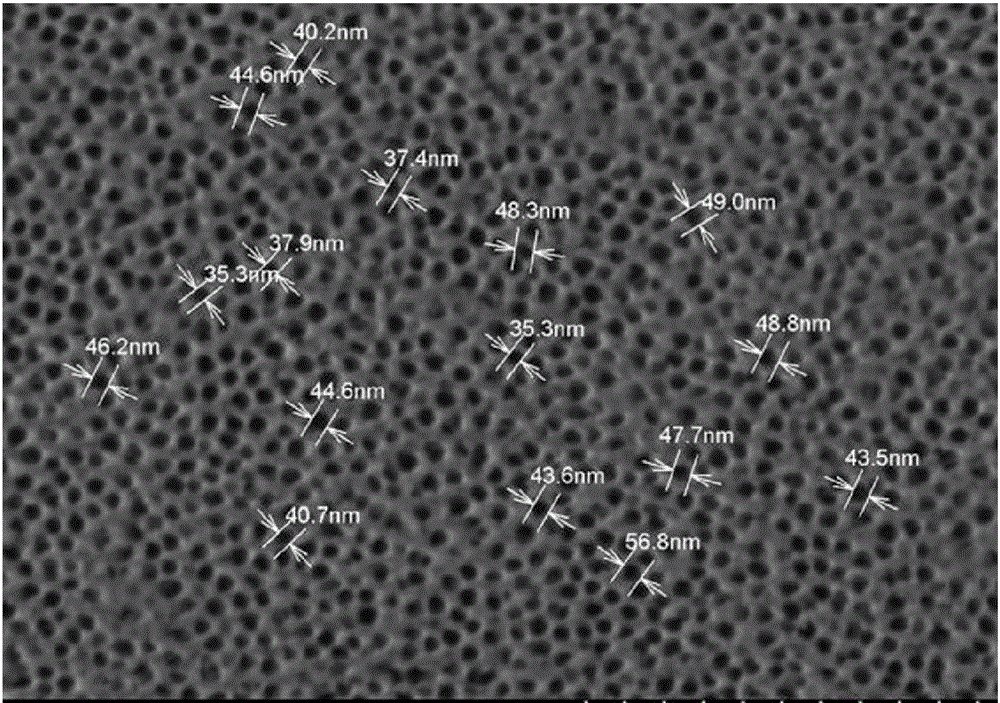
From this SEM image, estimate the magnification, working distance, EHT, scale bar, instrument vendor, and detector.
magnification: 50 K X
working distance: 6.5 mm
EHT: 2 kV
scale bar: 1000 nm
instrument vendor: Hitachi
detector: SE(M,LA0)